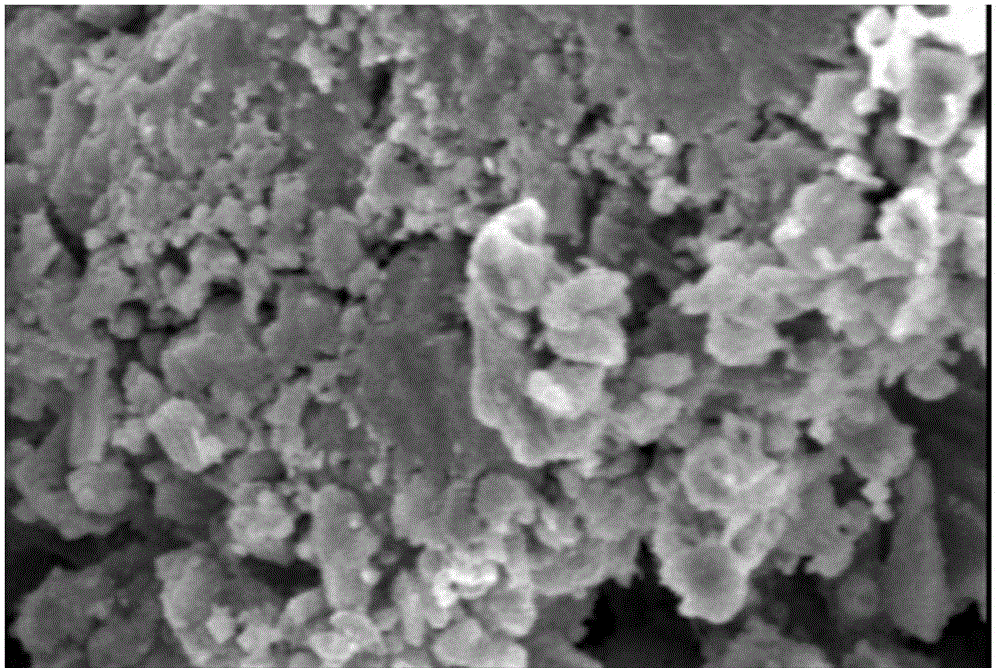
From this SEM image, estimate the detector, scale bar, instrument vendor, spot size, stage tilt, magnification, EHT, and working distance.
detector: SEI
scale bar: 2000 nm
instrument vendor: JEOL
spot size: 50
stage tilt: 0°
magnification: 5.5 K X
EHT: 10 kV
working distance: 13 mm